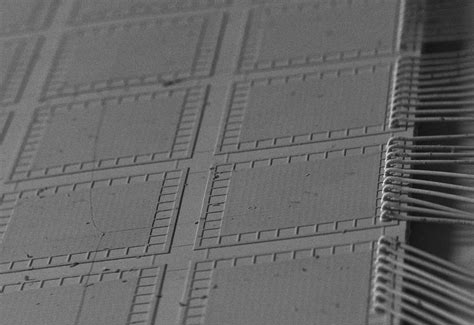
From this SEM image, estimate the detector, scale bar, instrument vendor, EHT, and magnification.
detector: SEC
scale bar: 1000000 nm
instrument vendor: JEOL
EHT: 20 kV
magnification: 0.05 K X